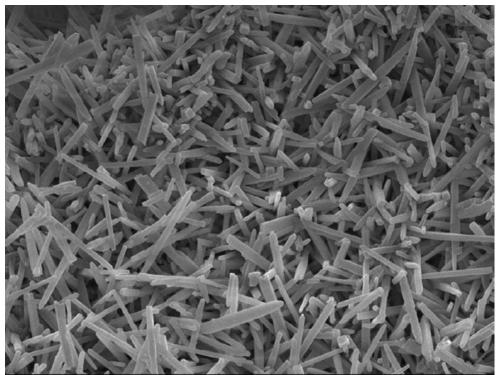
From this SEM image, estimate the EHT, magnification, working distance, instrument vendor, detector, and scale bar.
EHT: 10 kV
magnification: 5 K X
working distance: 9.9 mm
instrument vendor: JEOL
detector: SEI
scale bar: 1000 nm